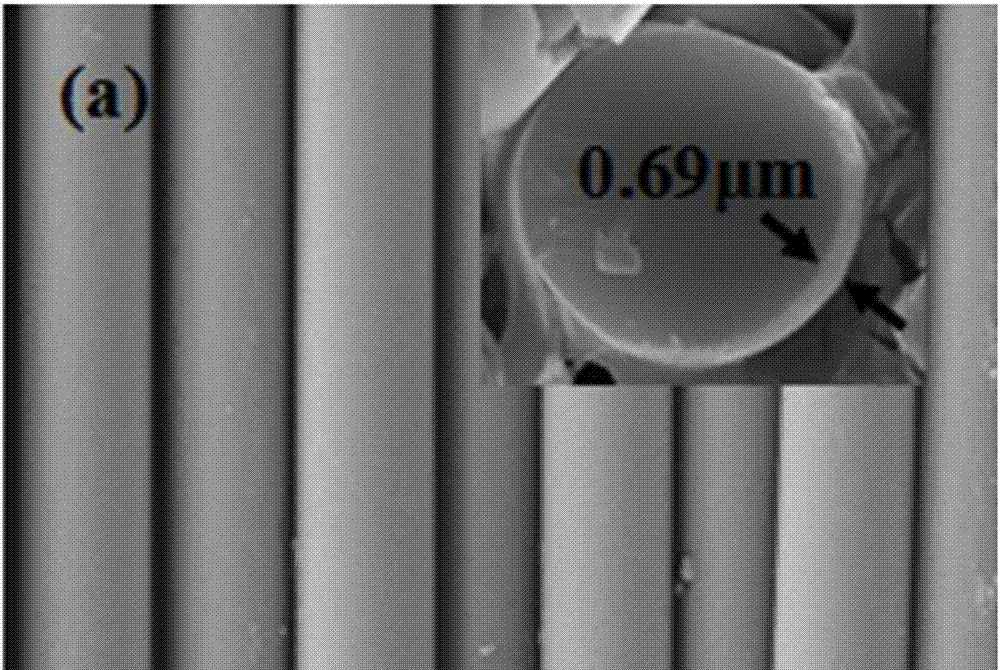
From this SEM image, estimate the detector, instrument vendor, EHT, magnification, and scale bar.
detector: SEI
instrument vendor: JEOL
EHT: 20 kV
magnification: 2 K X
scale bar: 10000 nm